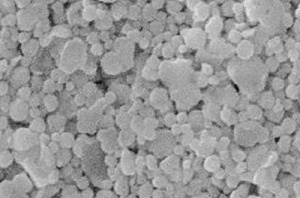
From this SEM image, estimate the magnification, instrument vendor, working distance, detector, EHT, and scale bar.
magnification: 10 K X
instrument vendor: FEI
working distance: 9.7 mm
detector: ETD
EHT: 20 kV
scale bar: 100 nm